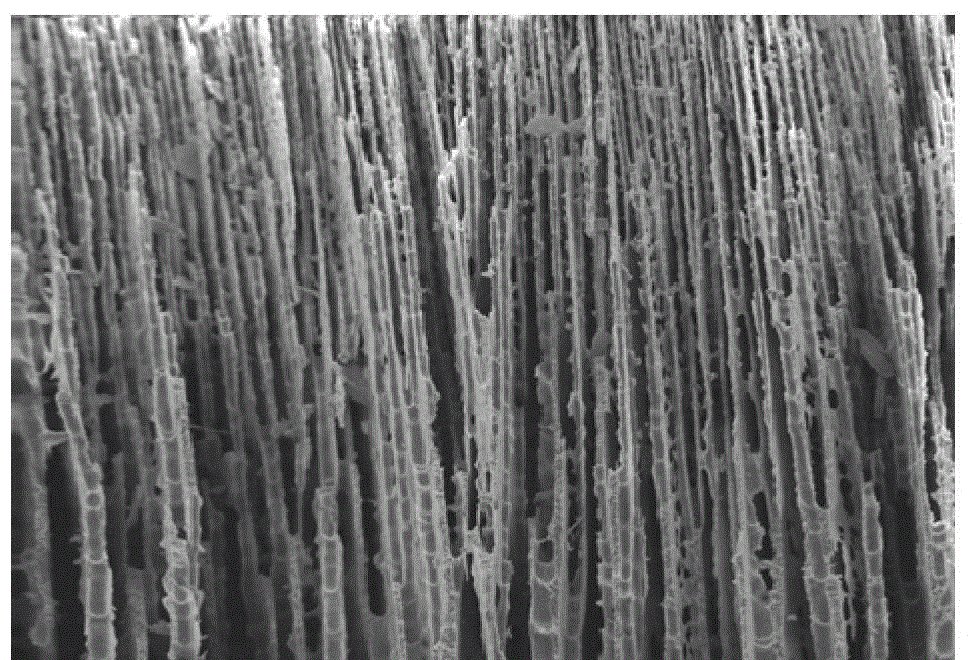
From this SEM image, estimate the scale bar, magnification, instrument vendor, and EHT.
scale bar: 100000 nm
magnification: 0.2 K X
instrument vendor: other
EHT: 25 kV